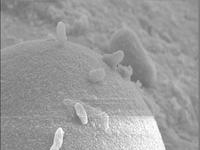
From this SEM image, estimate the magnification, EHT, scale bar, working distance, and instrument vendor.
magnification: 6 K X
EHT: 9 kV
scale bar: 1000 nm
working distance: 12 mm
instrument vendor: JEOL